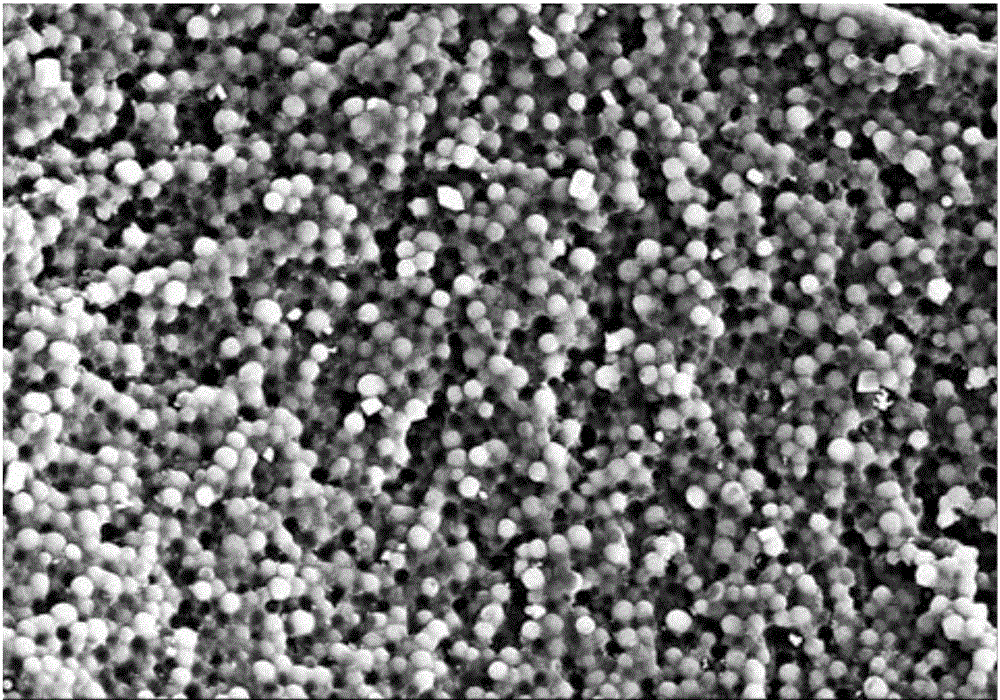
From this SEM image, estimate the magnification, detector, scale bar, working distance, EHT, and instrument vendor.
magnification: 1 K X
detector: SE(M)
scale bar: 50000 nm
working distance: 14.9 mm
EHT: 15 kV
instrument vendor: Hitachi